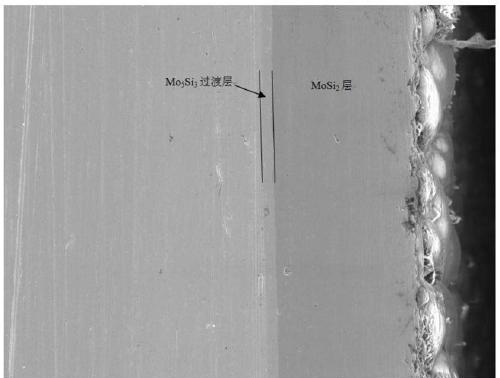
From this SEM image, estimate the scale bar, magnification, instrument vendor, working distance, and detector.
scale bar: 50000 nm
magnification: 1 K X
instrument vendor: Tescan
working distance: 18.54 mm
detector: SE